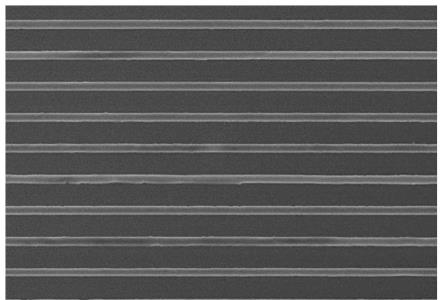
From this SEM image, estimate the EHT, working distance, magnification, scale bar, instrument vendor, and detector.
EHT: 10 kV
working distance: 9.6 mm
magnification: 1.3 K X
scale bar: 40000 nm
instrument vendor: Hitachi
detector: SE(UL)